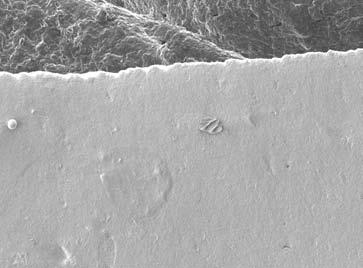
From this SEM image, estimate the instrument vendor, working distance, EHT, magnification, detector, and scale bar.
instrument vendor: JEOL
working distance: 8 mm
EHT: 5 kV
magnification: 0.04 K X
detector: LEI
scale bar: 100000 nm